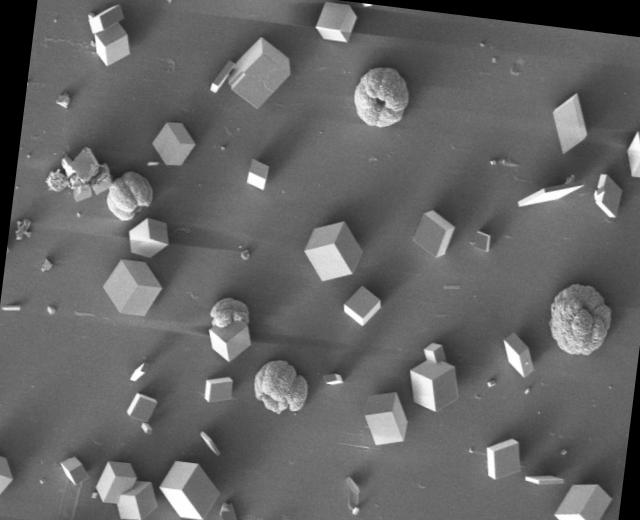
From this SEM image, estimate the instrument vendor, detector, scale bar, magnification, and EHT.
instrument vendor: Tescan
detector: SE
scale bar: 200000 nm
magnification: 0.4 K X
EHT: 5 kV